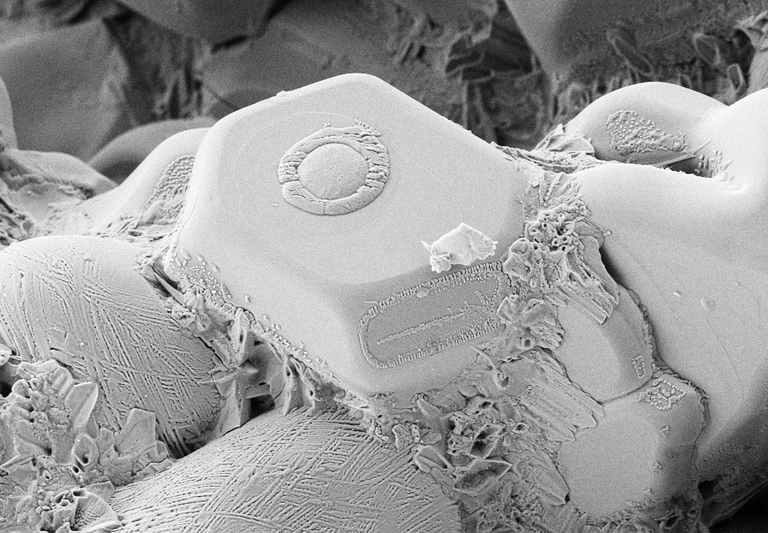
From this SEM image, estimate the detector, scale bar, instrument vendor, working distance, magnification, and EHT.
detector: SE2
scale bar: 2000 nm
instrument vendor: Zeiss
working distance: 12 mm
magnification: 1.5 K X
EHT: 5 kV